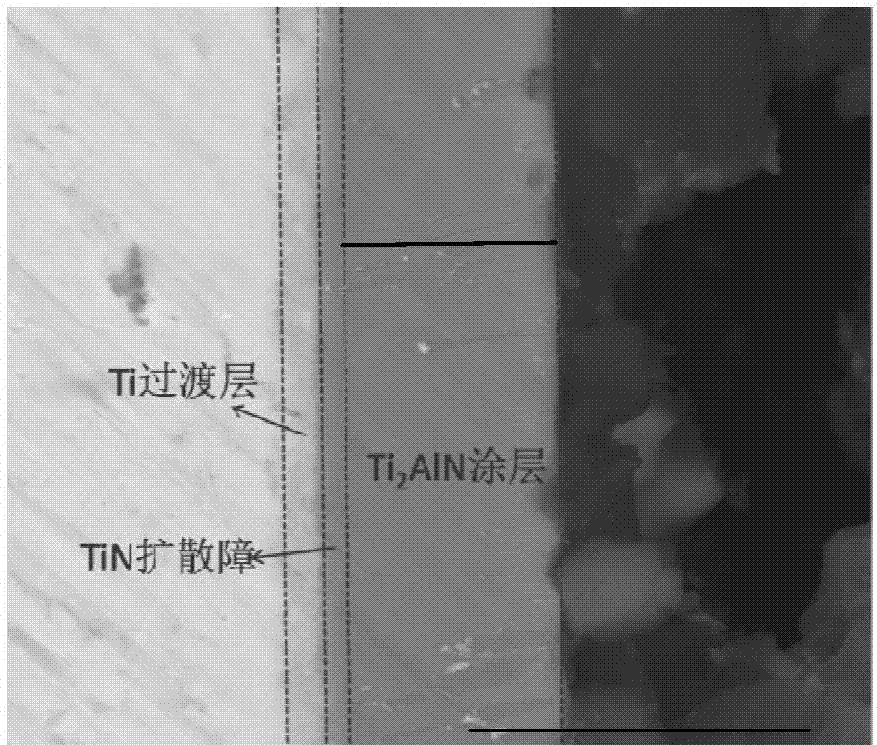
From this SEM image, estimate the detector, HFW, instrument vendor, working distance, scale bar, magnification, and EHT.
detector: vCD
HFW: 25.4 µm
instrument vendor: FEI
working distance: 9.8 mm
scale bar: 10000 nm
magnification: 5 K X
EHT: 20 kV